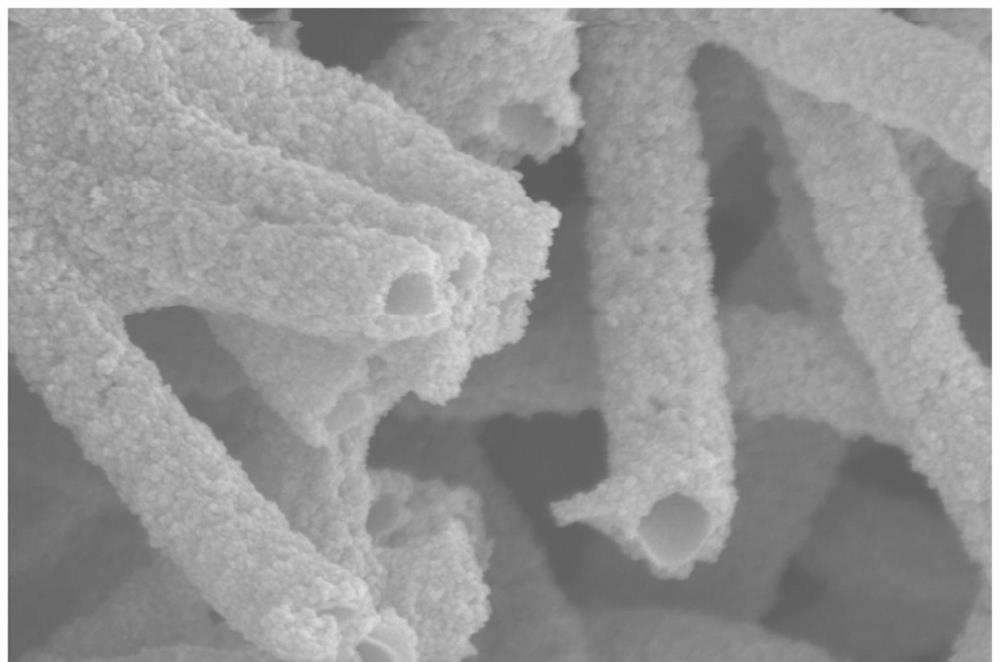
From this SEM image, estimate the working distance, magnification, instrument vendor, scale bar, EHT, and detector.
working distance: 7.9 mm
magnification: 70 K X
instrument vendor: Zeiss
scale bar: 100 nm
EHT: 5 kV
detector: InLens